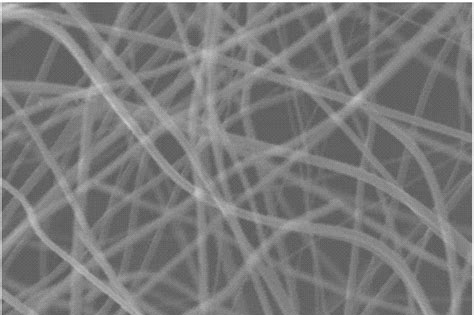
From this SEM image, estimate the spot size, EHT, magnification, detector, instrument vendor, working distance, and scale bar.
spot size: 41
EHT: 30 kV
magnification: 16 K X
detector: SEI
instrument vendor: JEOL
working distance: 10 mm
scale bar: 1000 nm